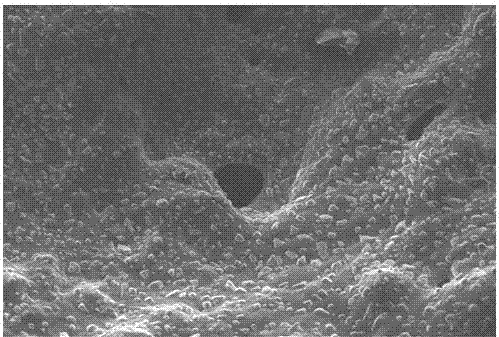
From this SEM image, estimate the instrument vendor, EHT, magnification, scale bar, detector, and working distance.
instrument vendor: Zeiss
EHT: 8 kV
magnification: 0.695 K X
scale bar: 20000 nm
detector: SE2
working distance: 9.8 mm